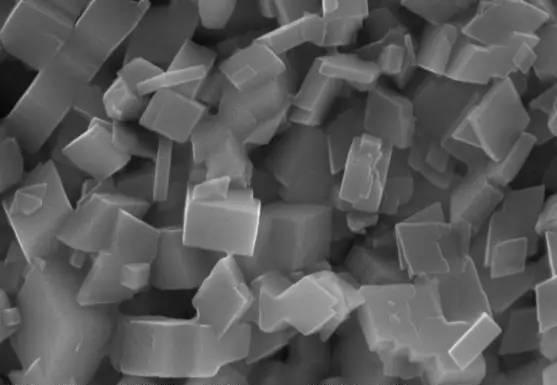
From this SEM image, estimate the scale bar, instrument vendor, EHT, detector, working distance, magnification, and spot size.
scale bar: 1000 nm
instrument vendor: JEOL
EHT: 20 kV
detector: SEI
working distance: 9 mm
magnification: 20 K X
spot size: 30